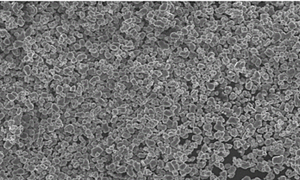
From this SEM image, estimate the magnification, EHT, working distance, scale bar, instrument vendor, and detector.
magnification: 1 K X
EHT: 15 kV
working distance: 8.3 mm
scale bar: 50000 nm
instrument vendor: Hitachi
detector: SE(U)